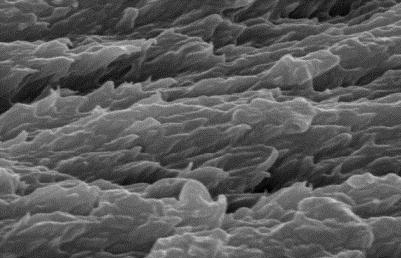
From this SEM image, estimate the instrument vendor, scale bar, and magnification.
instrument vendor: JEOL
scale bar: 1000 nm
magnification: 10 K X